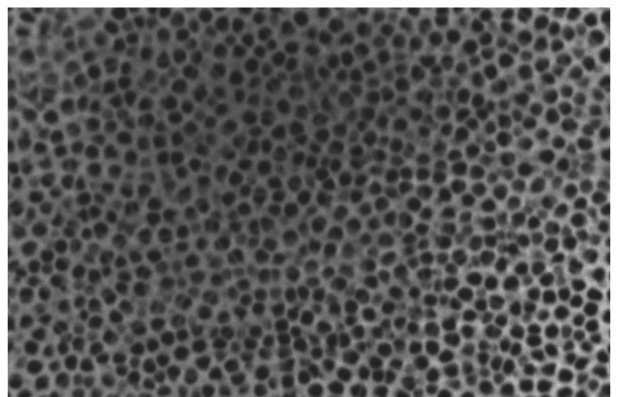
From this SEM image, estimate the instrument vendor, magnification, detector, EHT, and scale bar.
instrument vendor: JEOL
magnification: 10 K X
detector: SEI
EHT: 15 kV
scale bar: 1000 nm